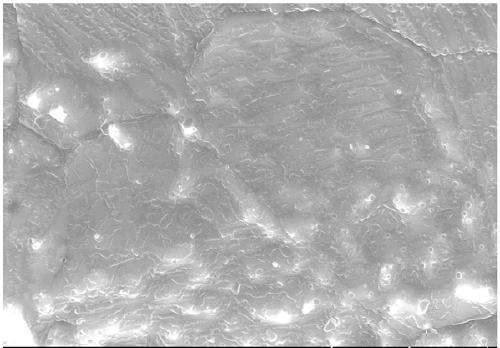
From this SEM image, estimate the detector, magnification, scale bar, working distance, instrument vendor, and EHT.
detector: SE(U)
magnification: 20 K X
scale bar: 2000 nm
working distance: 10.6 mm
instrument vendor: Hitachi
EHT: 15 kV